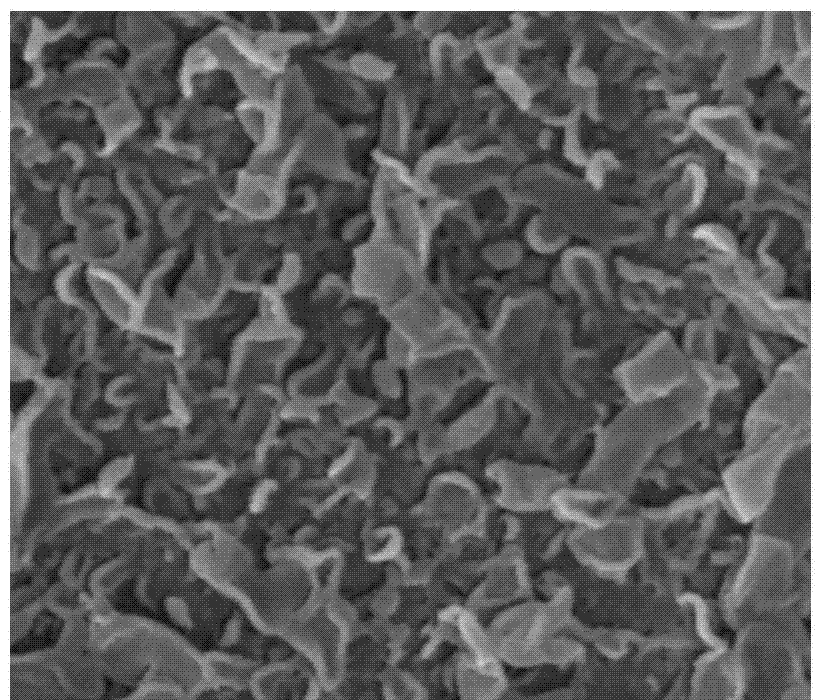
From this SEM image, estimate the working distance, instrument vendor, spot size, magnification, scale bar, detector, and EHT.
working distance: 5 mm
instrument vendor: FEI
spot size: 3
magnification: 80 K X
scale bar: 500 nm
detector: ETD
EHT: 10 kV